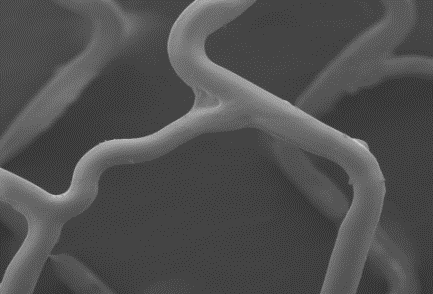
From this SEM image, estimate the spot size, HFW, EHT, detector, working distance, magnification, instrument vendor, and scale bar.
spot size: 3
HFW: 942 µm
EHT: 1 kV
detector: ETD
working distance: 8.6 mm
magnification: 0.317 K X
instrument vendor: FEI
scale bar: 300000 nm